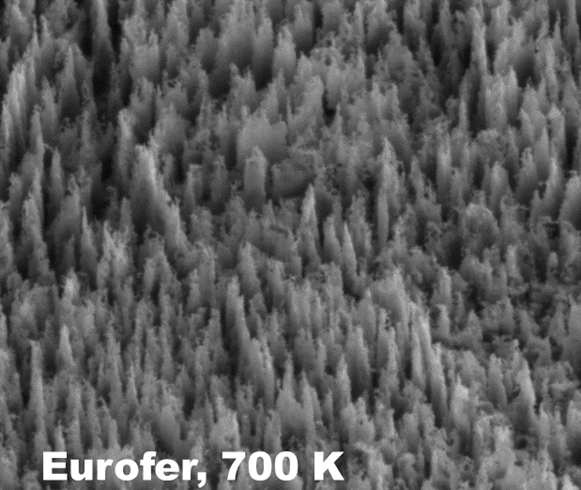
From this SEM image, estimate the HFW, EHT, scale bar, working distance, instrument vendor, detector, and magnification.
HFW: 5.12 µm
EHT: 5 kV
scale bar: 1000 nm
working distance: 4.1 mm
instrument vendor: FEI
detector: ETD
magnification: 25 K X